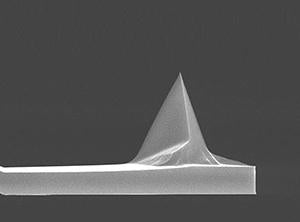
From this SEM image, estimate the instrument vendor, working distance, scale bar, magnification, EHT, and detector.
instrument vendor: FEI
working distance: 4.2 mm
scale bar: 10000 nm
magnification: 3.5 K X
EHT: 15 kV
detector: TLD